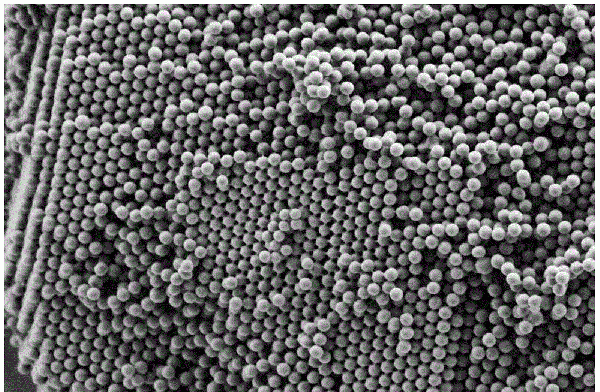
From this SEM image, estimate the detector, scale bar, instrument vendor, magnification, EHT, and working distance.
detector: SE2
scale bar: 1000 nm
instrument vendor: Zeiss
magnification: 10 K X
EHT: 1 kV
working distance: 4.7 mm